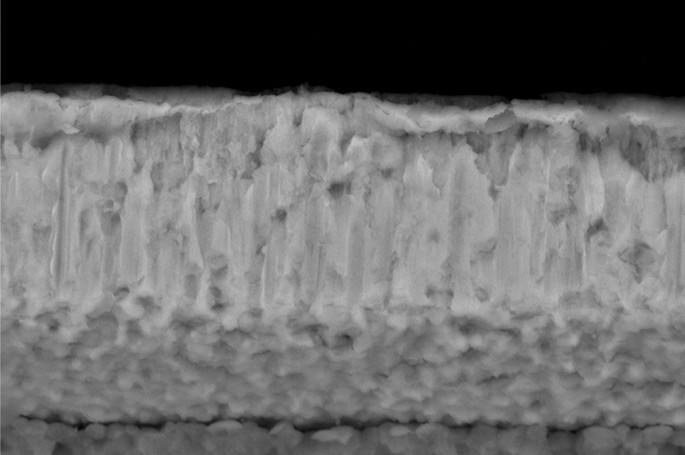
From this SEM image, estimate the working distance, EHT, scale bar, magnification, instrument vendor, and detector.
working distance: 8.2 mm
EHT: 15 kV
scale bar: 1000 nm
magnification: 30 K X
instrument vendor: Zeiss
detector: NTS BSD